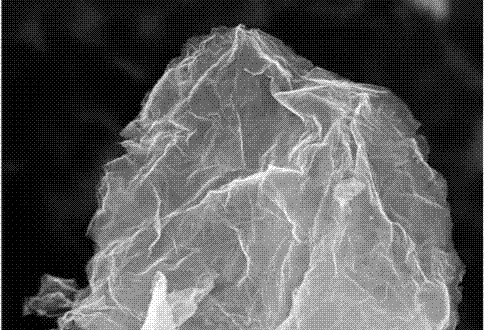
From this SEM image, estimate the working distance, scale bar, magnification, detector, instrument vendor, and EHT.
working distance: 8.5 mm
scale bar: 200 nm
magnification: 20 K X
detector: InLens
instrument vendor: Zeiss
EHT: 3 kV